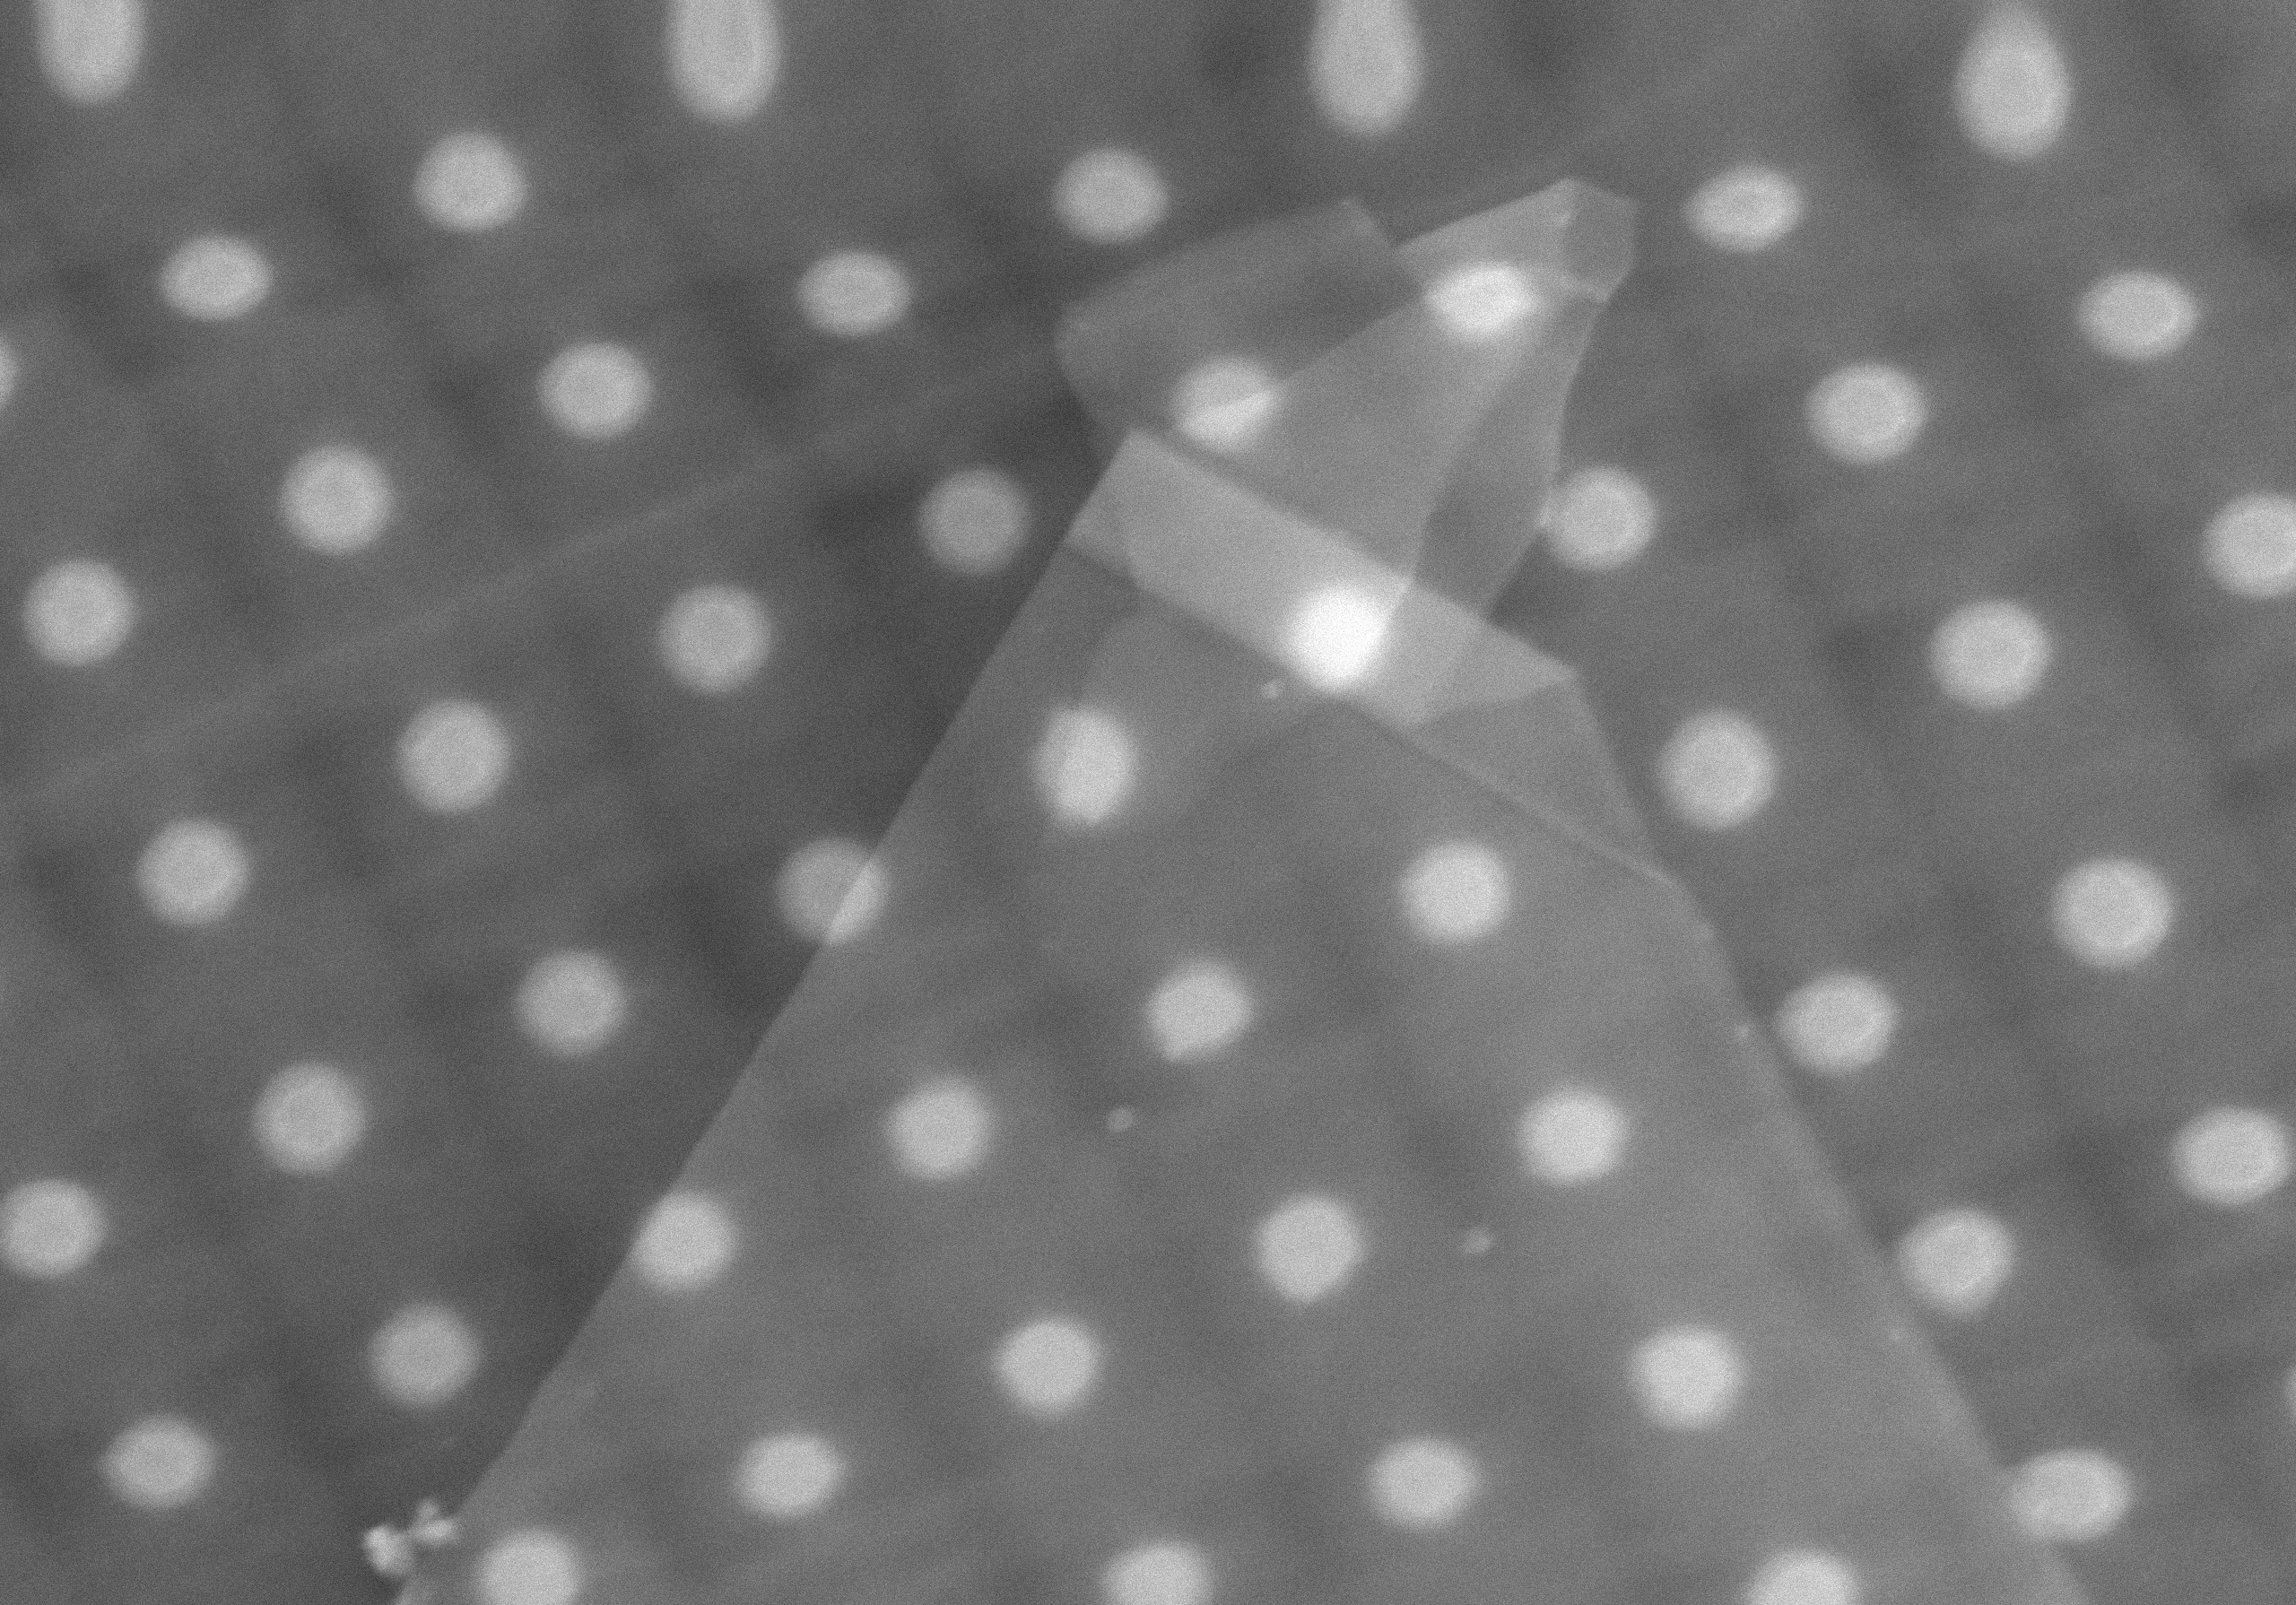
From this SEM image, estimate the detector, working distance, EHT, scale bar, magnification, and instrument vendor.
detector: LD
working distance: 6.2 mm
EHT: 15 kV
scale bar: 2000 nm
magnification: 22 K X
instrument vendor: Hitachi